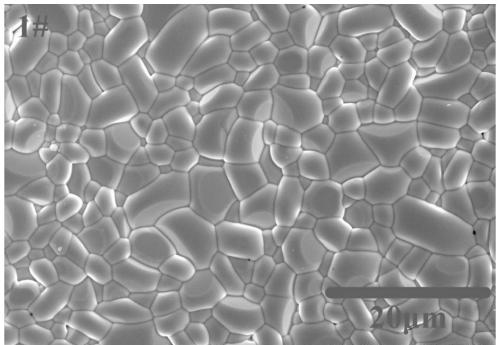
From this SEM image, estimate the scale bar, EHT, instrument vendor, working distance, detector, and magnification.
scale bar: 20000 nm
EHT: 15 kV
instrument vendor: Hitachi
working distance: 9.5 mm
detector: SE(M)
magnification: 2 K X